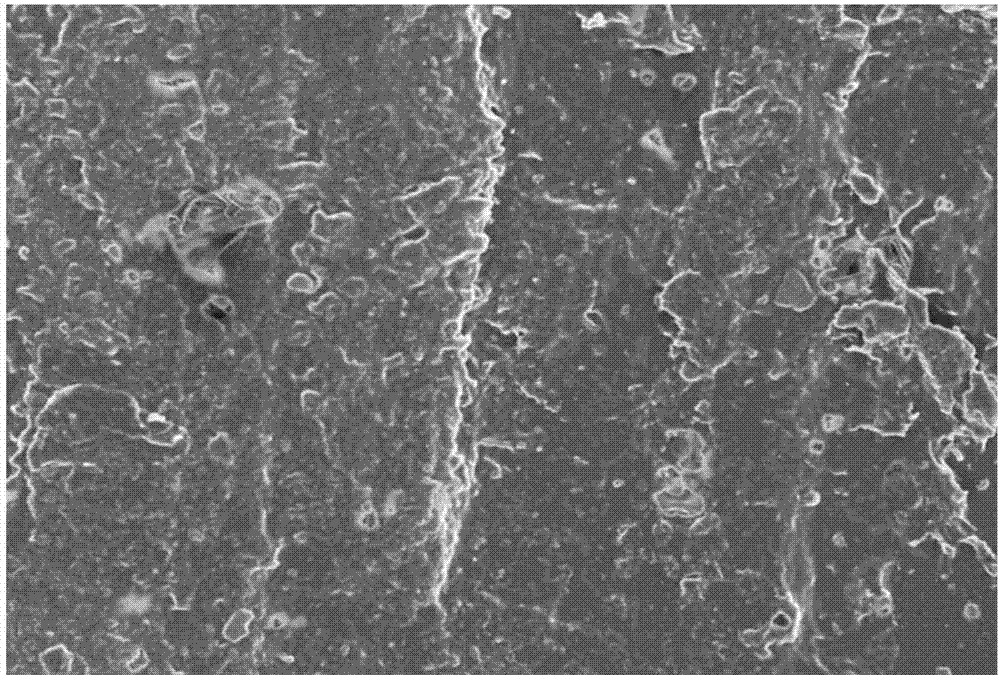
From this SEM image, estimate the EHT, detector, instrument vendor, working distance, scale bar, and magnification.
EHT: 5 kV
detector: SE(M)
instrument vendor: Hitachi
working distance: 9 mm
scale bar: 50000 nm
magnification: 1 K X